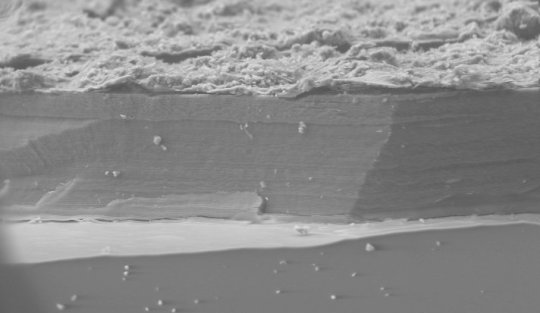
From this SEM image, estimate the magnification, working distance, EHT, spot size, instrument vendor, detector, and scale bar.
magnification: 1 K X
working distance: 10.7 mm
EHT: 15 kV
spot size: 4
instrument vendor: FEI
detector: GSE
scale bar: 50000 nm